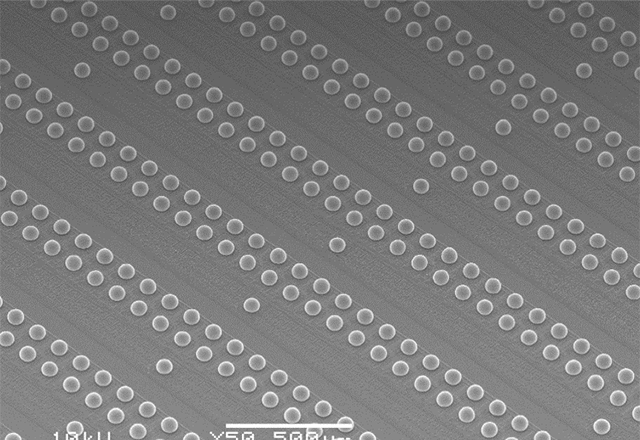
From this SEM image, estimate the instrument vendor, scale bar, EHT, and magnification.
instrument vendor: JEOL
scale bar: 500000 nm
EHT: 10 kV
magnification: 0.05 K X